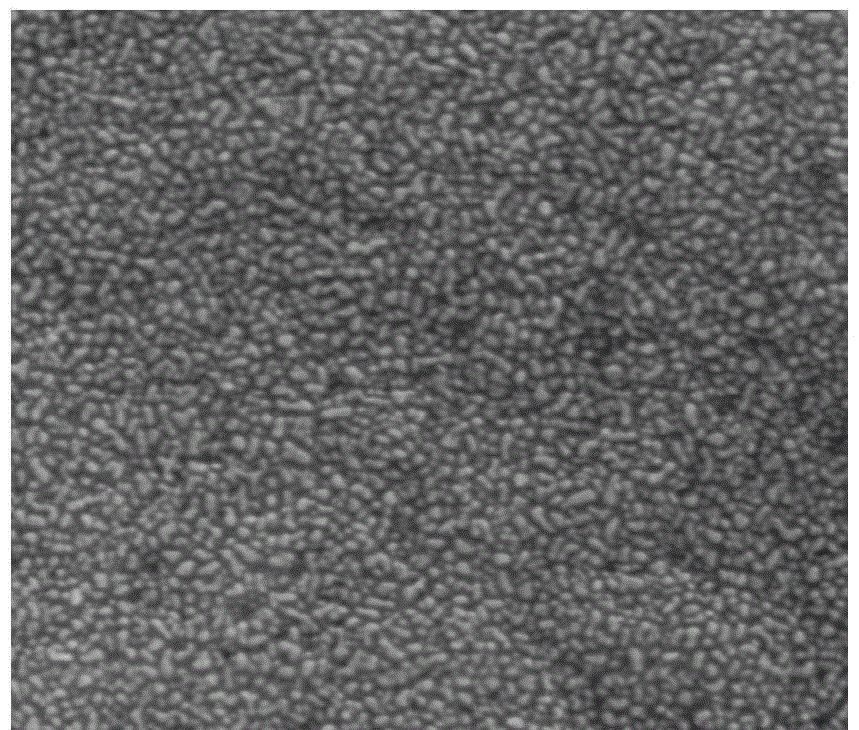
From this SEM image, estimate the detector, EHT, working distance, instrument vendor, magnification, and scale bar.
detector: ETD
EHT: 20 kV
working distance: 9.3 mm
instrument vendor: FEI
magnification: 200 K X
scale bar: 500 nm